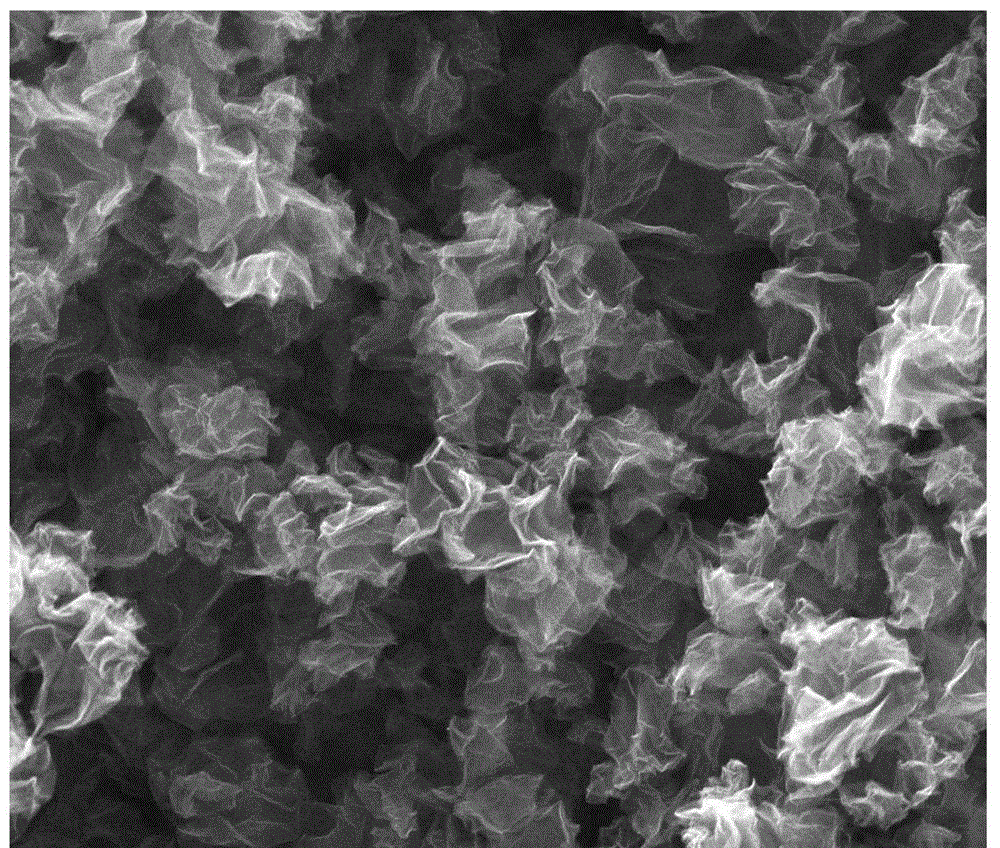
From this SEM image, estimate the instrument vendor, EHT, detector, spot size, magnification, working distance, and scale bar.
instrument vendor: FEI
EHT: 20 kV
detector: ETD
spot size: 3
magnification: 60 K X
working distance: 10 mm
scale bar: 2000 nm